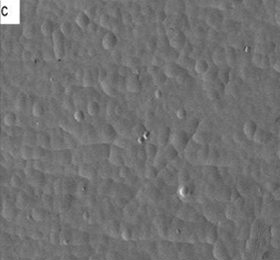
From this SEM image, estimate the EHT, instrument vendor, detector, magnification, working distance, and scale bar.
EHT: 5 kV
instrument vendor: JEOL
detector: LEI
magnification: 0.5 K X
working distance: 8.3 mm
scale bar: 10000 nm